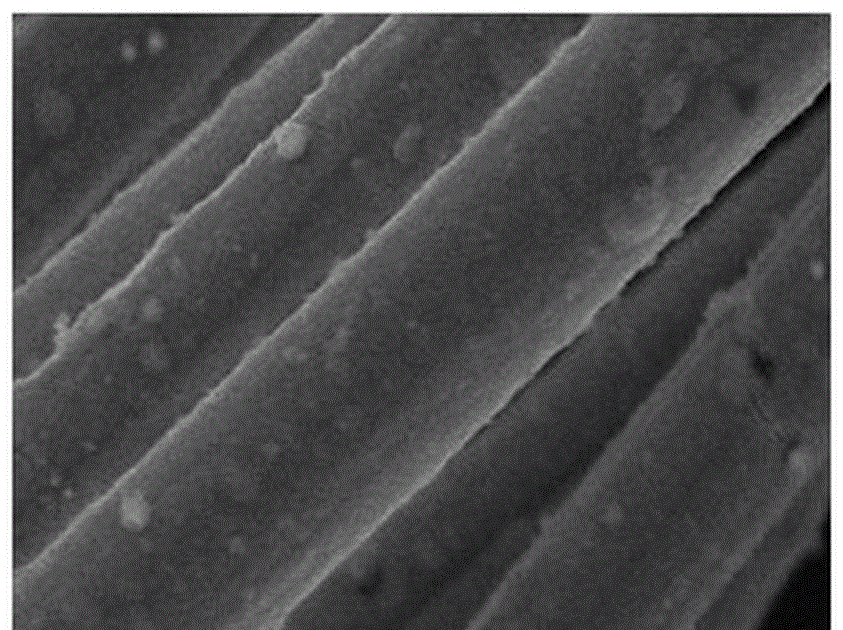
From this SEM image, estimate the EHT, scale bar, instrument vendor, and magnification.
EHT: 25 kV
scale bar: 5000 nm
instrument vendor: JEOL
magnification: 3 K X